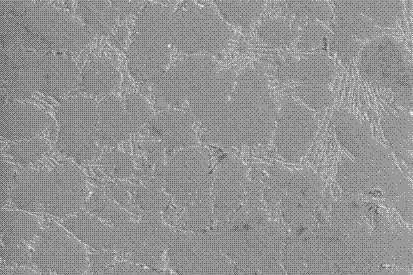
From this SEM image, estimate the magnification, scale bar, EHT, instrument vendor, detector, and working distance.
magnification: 0.5 K X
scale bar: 10000 nm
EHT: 20 kV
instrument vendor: Zeiss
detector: SE1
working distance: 8 mm